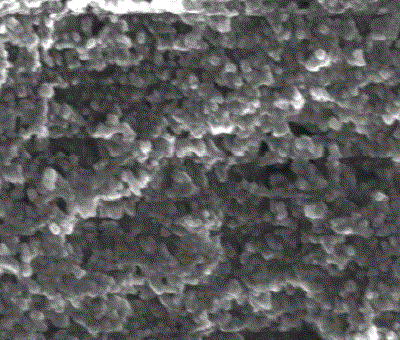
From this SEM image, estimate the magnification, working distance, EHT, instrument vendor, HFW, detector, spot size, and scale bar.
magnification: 100 K X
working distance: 5.6 mm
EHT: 15 kV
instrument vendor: FEI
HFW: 2.98 µm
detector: TLD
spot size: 4.5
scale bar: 500 nm